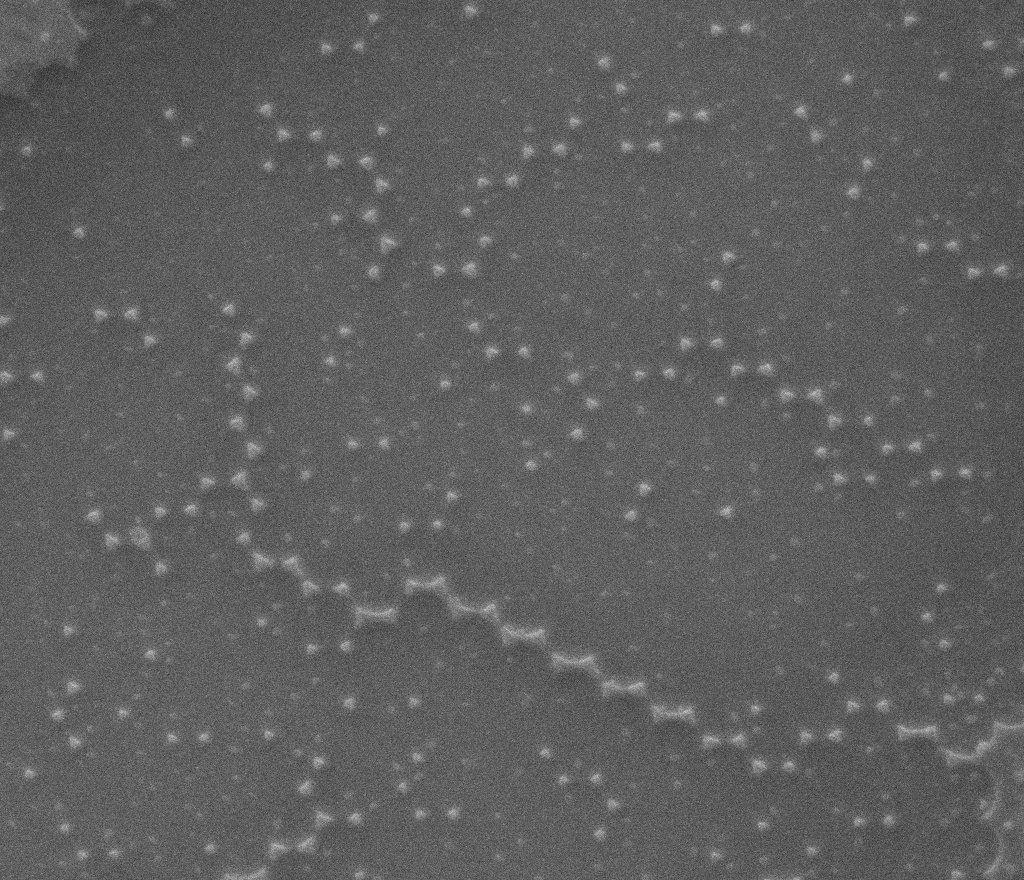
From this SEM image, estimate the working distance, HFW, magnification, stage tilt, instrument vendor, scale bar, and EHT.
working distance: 12 mm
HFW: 9.11 µm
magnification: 29.679 K X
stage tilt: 0°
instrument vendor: FEI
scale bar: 3000 nm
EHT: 25 kV